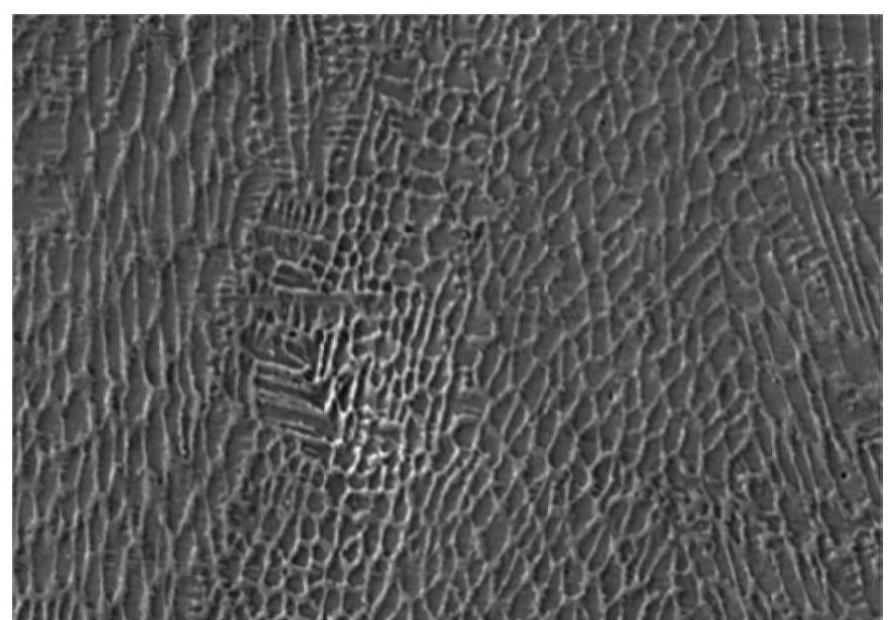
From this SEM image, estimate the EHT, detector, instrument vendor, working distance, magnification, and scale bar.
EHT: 10 kV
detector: LM(UL)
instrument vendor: Hitachi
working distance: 9.1 mm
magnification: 2 K X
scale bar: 20000 nm